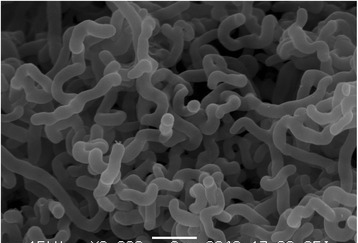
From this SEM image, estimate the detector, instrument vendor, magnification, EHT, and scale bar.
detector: SEI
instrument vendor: JEOL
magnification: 8 K X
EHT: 15 kV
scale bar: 2000 nm